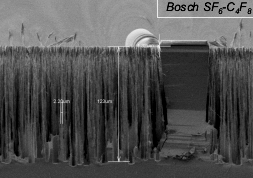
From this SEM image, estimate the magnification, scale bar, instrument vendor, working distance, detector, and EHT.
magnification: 0.45 K X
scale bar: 100000 nm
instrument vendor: Hitachi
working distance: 8.2 mm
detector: SE(M)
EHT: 1.5 kV